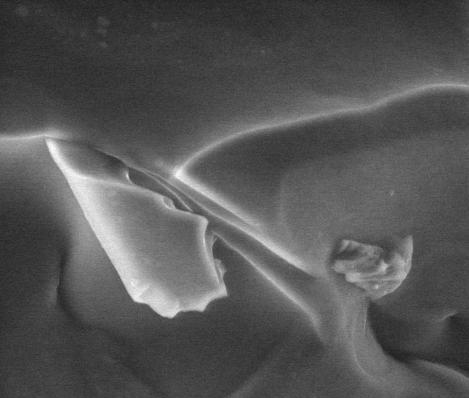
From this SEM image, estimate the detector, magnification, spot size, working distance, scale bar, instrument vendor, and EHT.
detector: GSED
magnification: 2.5 K X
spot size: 3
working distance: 10.4 mm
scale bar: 50000 nm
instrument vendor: FEI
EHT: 15 kV